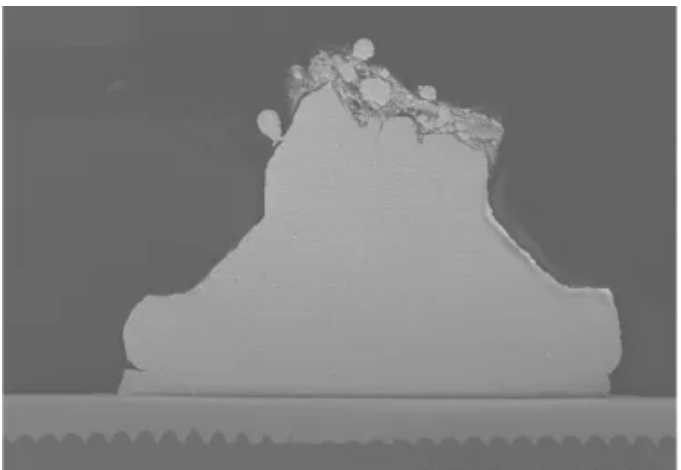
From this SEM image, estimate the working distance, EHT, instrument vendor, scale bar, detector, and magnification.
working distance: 8.5 mm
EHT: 10 kV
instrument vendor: Hitachi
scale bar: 30000 nm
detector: SE(L)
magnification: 1.5 K X